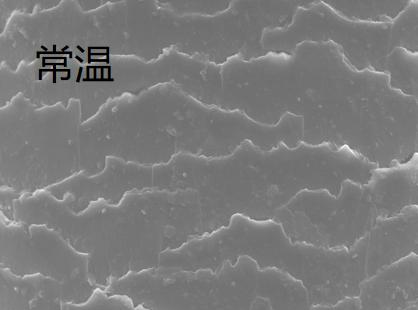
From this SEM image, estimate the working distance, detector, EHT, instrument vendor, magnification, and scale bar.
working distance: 6.55 mm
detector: In-Beam SE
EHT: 10 kV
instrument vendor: Tescan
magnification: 5 K X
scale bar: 10000 nm